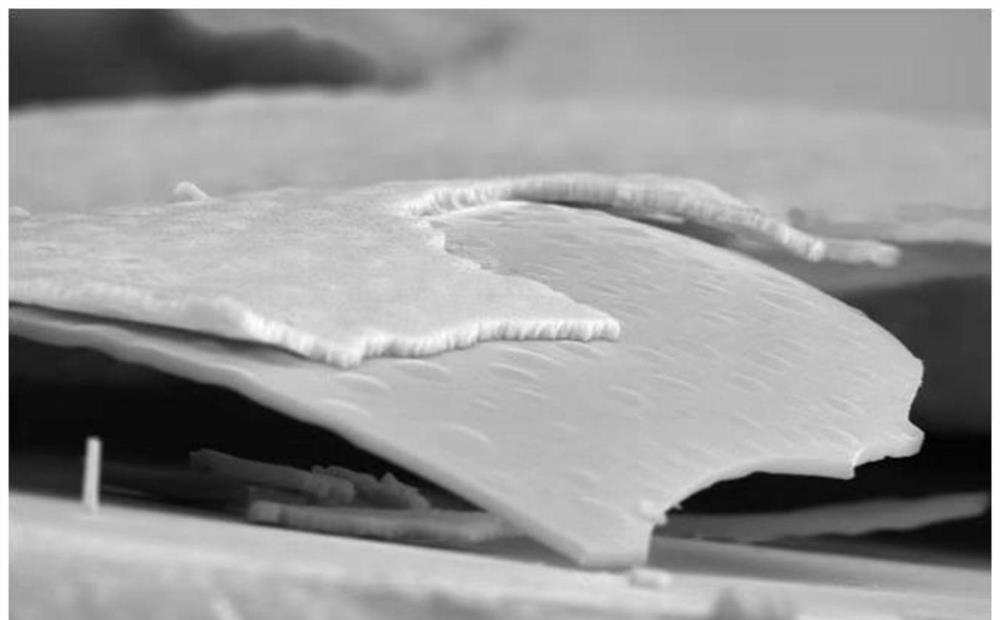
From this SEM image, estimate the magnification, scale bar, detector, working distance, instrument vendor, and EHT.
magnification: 6 K X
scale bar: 5000 nm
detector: SE(M)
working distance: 14.6 mm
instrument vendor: Hitachi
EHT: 15 kV